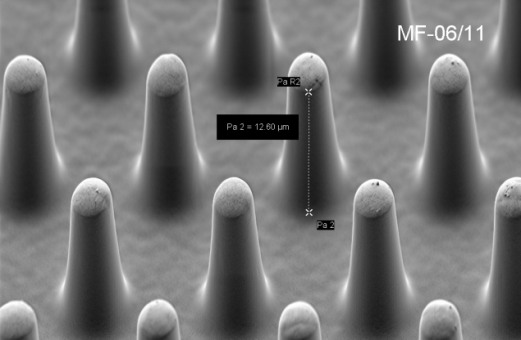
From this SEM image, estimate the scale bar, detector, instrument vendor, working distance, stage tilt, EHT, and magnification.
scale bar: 2000 nm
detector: SE2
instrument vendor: Zeiss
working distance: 7.2 mm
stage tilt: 45°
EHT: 2 kV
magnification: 5 K X